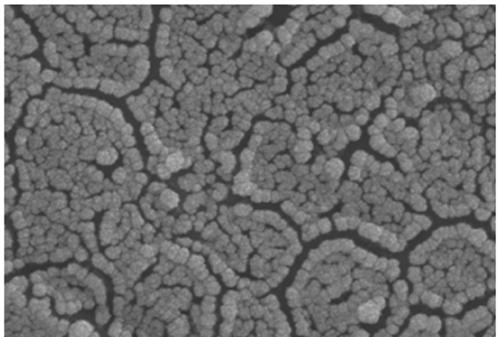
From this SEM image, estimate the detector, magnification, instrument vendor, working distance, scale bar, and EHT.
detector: SE2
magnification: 20 K X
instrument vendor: Zeiss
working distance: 12.7 mm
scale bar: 200 nm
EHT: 15 kV